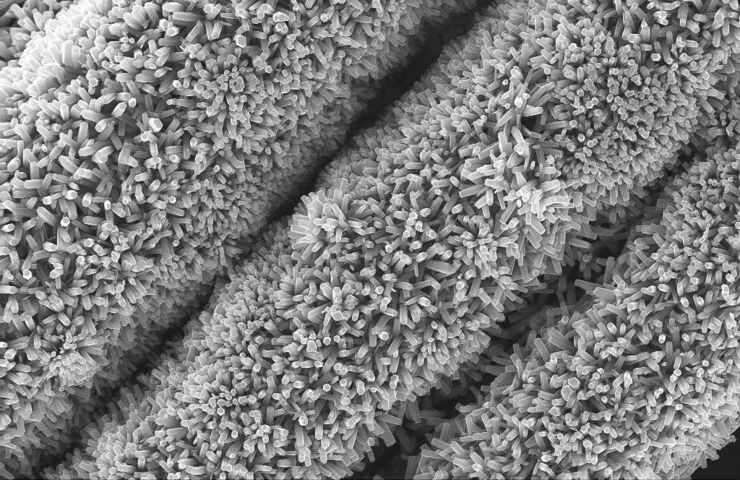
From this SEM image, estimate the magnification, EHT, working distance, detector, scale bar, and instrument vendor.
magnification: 5.56 K X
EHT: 20 kV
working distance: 9 mm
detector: InLens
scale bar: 2000 nm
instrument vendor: Zeiss